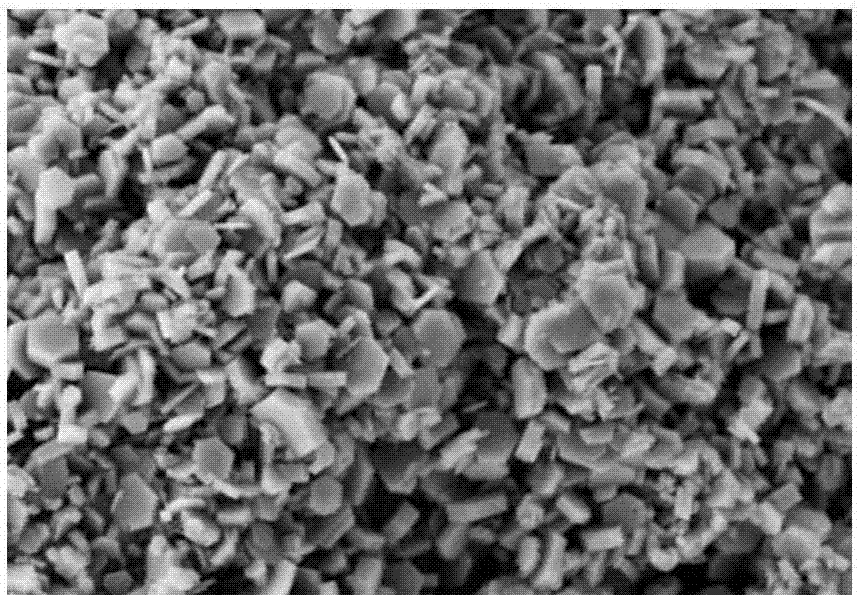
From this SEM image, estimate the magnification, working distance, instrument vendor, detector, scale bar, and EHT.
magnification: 20 K X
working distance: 7.1 mm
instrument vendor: Zeiss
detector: SE2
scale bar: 100 nm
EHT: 3 kV